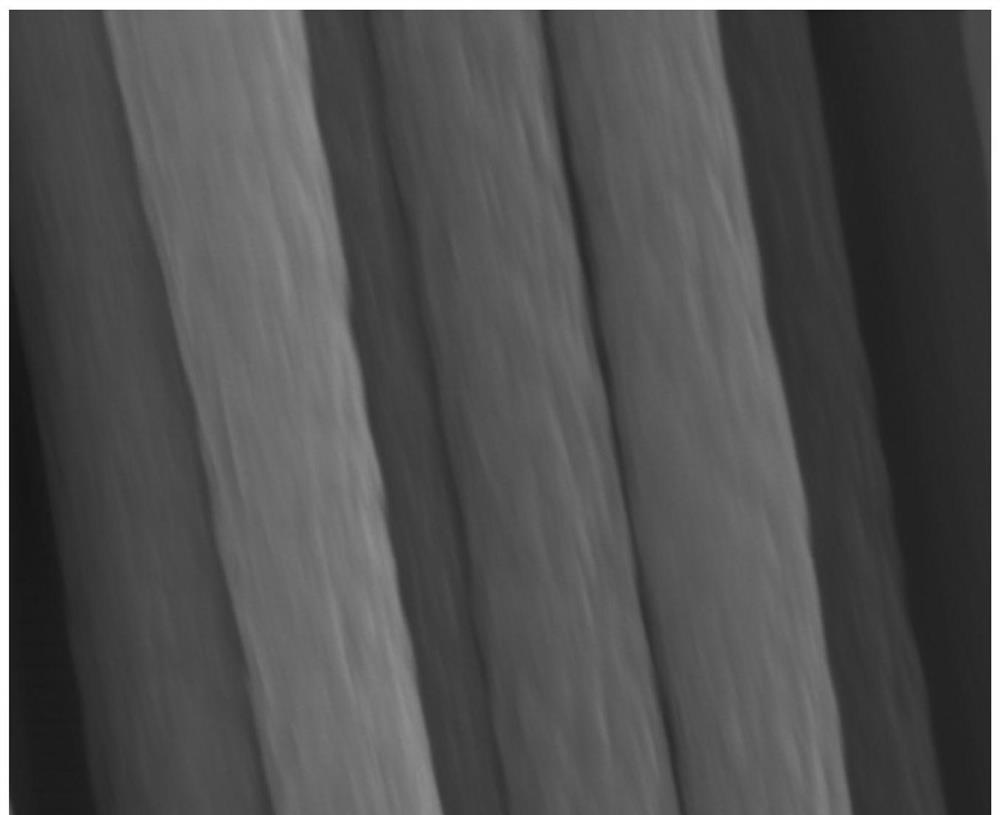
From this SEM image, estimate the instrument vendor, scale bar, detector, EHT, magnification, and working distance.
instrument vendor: Hitachi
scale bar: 1000 nm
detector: SE(M)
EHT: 10 kV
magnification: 40 K X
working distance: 18.2 mm